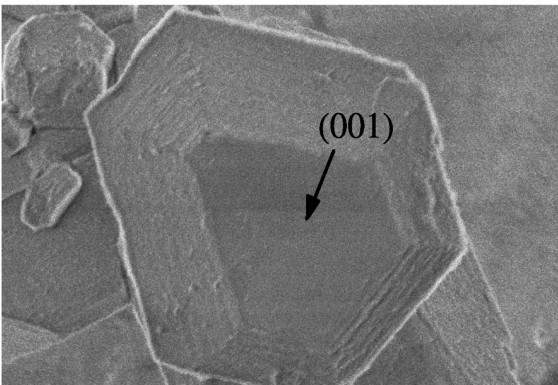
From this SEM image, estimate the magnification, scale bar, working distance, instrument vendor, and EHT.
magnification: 22 K X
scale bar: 1000 nm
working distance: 7 mm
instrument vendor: JEOL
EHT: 2 kV